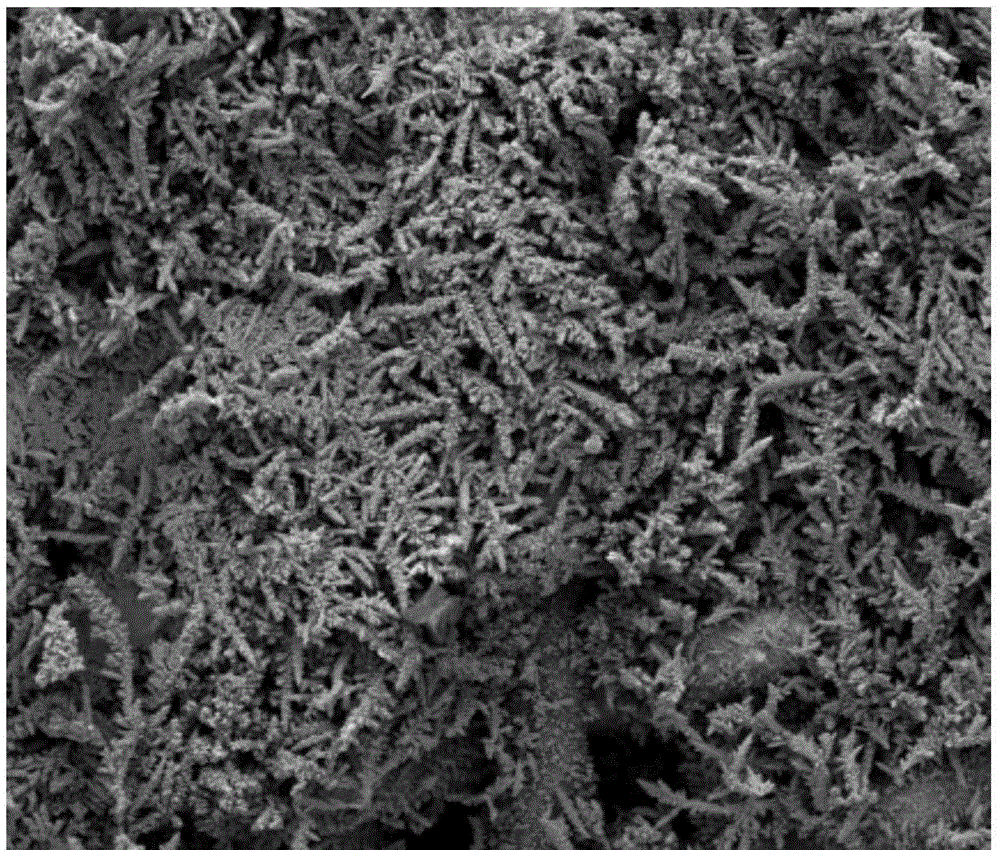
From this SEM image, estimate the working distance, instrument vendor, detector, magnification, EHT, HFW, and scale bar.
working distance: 10.6 mm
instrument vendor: FEI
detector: ETD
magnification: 0.2 K X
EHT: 5 kV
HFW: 635 µm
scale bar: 300000 nm